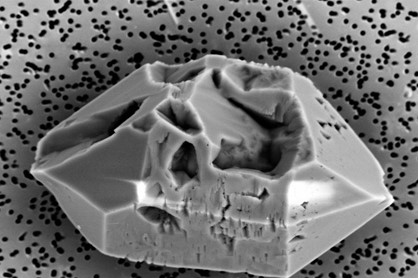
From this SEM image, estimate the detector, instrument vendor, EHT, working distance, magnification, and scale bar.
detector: NTS BSD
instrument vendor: Zeiss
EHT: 15 kV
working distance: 8.5 mm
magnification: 4 K X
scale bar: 10000 nm